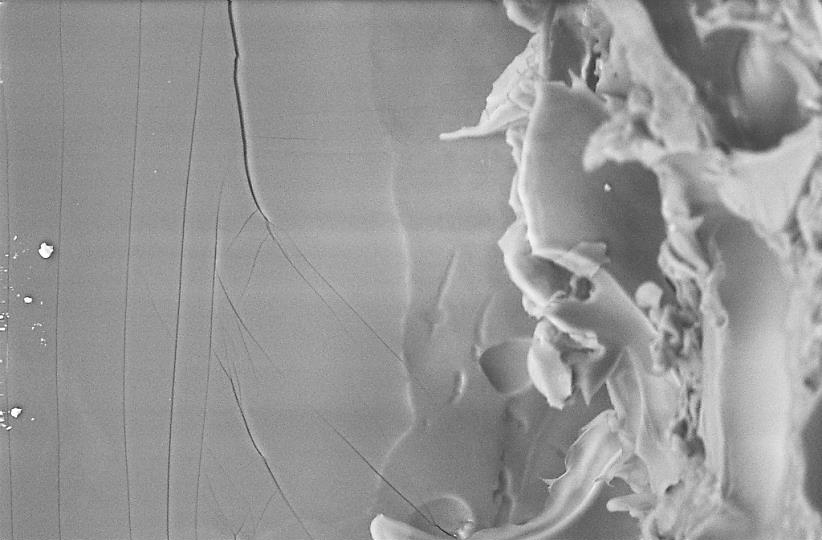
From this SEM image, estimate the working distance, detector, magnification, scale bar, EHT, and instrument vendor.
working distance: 15 mm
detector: BSD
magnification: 0.25 K X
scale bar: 20000 nm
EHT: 20 kV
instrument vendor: Zeiss